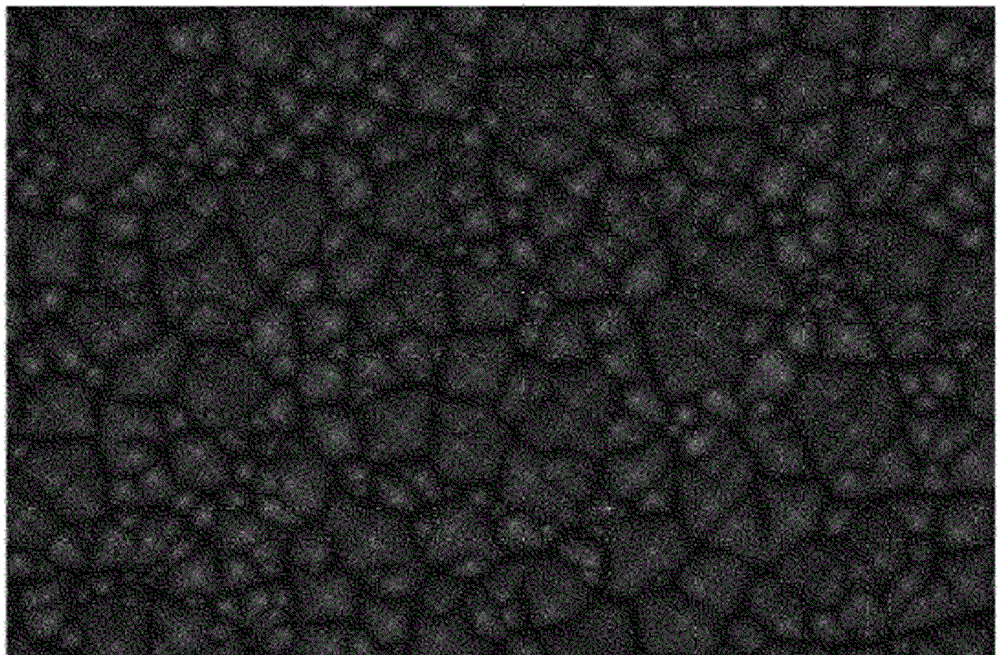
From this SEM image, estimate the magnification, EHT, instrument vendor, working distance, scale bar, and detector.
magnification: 2 K X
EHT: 15 kV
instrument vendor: Hitachi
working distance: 16.6 mm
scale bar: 20000 nm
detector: SE(U)